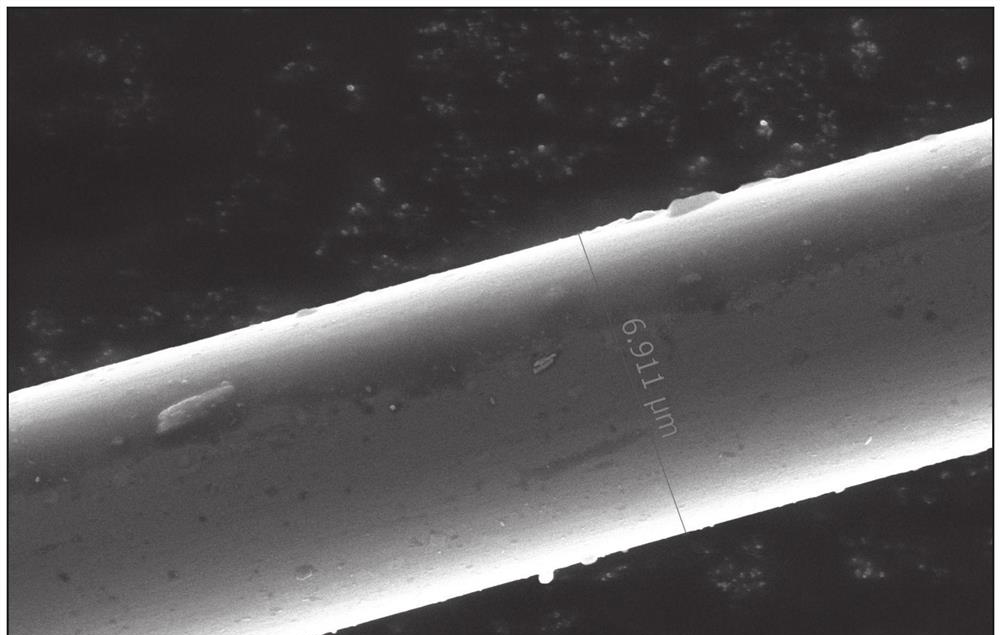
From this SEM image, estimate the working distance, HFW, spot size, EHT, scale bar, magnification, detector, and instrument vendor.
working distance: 17.1925 mm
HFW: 20.7 µm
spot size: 8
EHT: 10 kV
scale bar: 4000 nm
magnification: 20 K X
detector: T2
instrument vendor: FEI